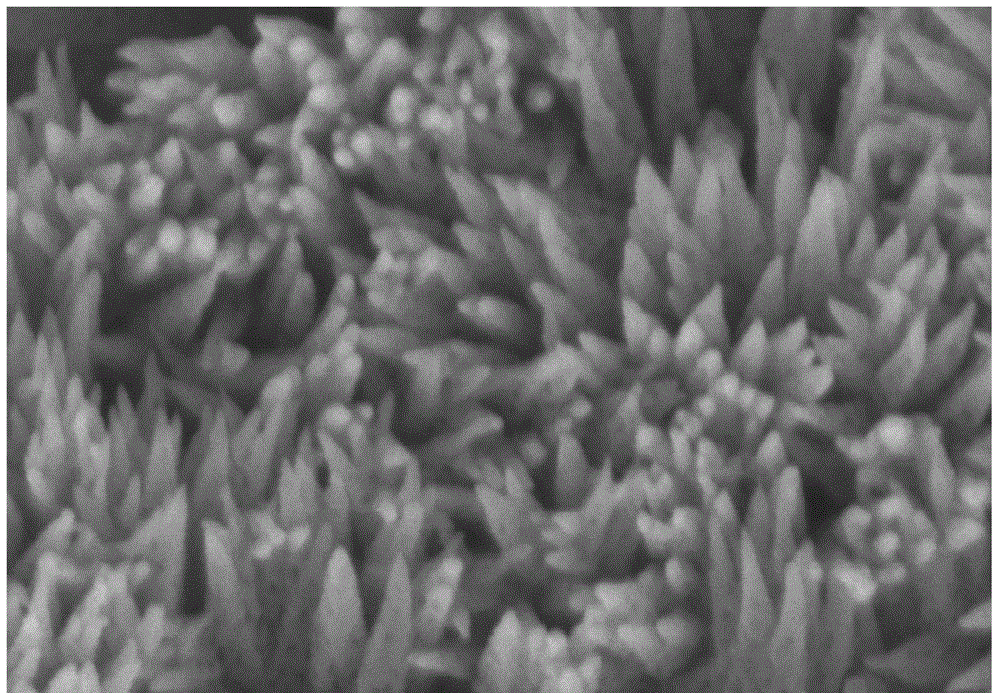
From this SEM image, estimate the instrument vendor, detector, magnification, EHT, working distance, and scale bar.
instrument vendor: Hitachi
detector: SE(U)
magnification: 100 K X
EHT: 5 kV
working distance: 10.9 mm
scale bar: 500 nm